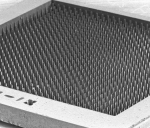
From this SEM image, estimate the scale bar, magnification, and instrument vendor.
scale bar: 1000000 nm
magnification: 0.019 K X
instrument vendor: JEOL